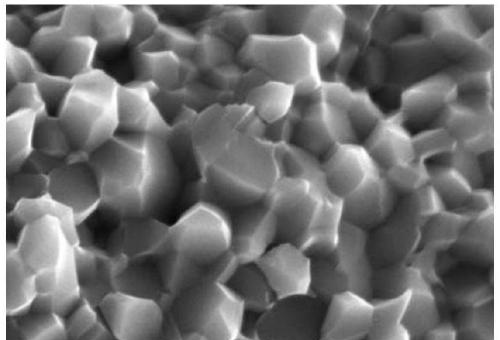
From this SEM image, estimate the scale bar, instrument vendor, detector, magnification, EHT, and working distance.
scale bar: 5000 nm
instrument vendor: Hitachi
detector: SE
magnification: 10 K X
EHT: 15 kV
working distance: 10.2 mm